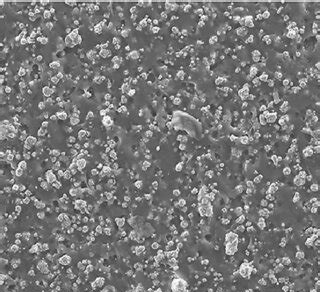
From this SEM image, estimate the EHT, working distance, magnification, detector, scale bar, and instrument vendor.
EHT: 20 kV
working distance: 10 mm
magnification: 0.35 K X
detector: LED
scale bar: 10000 nm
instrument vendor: JEOL